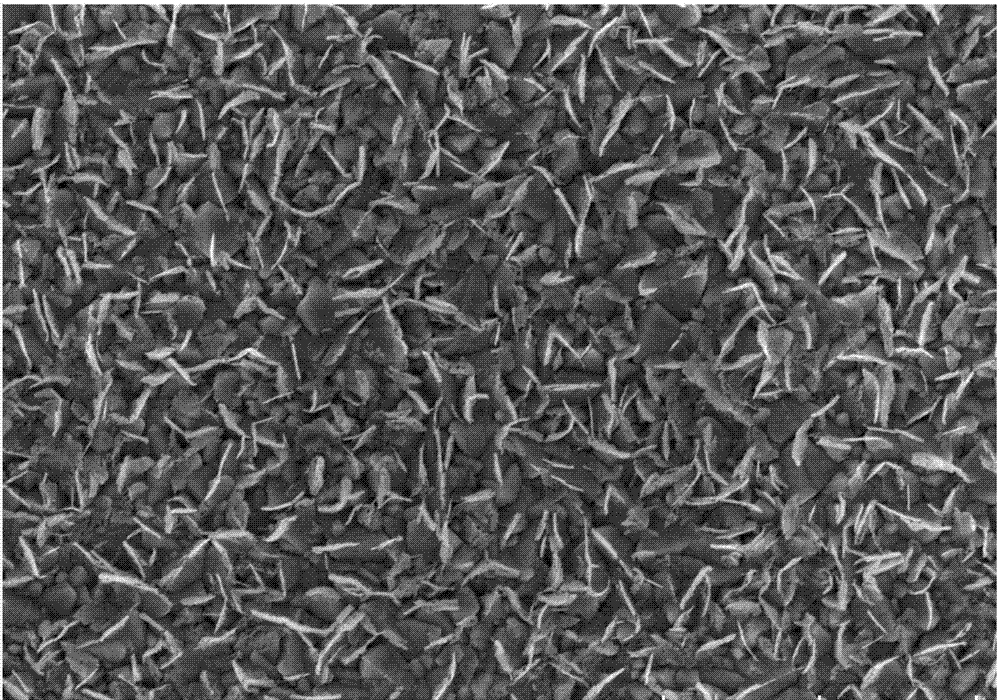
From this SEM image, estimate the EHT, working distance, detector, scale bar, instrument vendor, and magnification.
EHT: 5 kV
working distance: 3.6 mm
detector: SE(UL)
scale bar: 2000 nm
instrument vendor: Hitachi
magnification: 20 K X